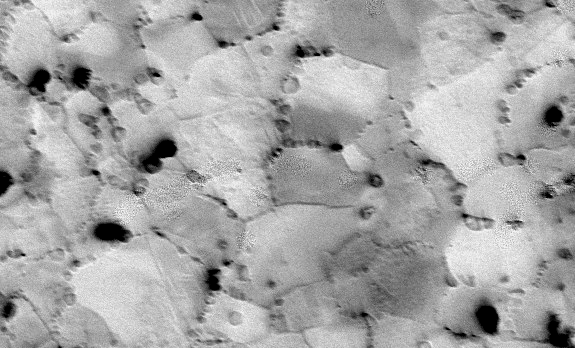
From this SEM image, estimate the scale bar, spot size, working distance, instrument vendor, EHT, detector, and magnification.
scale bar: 50000 nm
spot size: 6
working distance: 10.1 mm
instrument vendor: FEI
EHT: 20 kV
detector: BSE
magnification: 1 K X